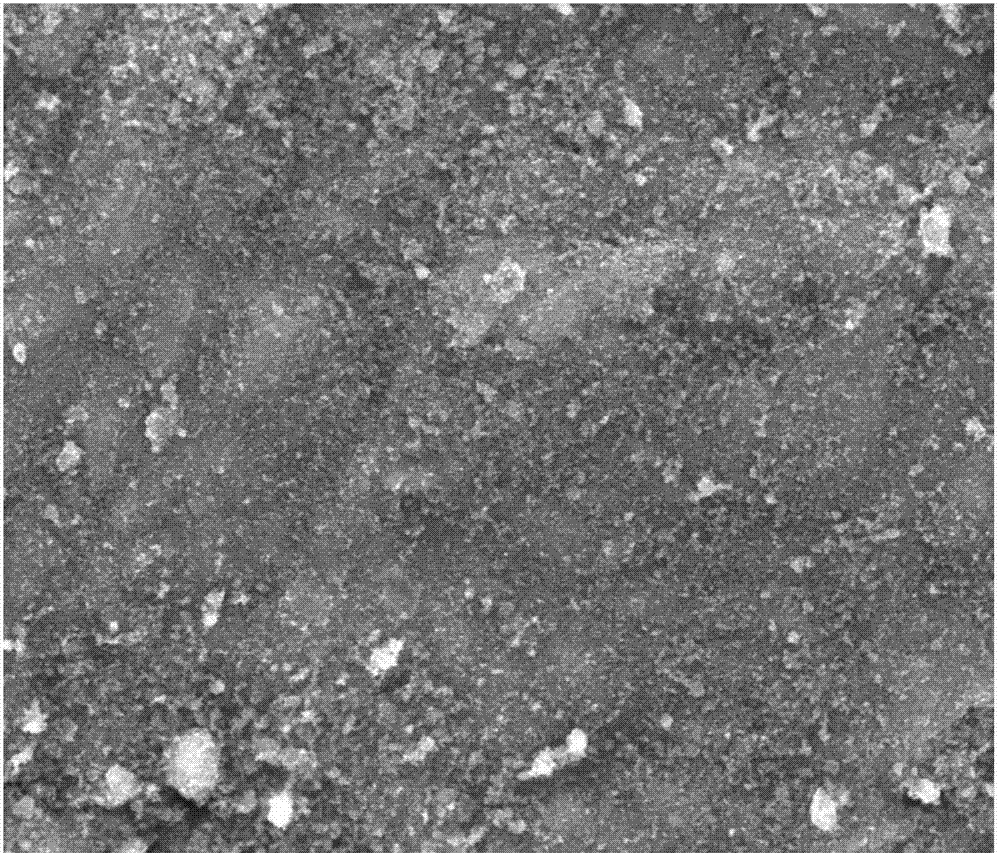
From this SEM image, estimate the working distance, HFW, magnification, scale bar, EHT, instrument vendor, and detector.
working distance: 10.6 mm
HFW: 63.5 µm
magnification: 2 K X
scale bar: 30000 nm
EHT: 20 kV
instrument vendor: FEI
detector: ETD